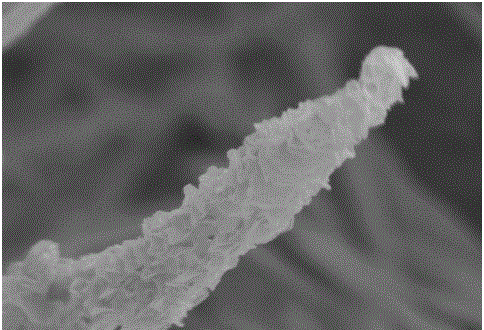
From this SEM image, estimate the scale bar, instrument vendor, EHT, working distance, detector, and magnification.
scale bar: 1000 nm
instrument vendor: Hitachi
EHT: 5 kV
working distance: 7.5 mm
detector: SE(M)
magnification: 40 K X